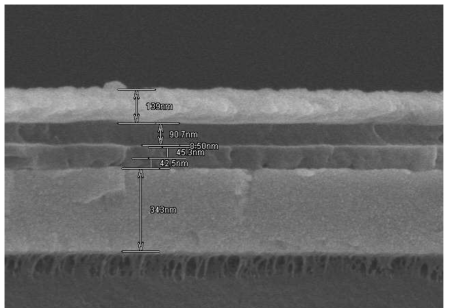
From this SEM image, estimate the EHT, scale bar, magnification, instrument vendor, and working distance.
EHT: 5 kV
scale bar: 500 nm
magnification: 70 K X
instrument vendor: Hitachi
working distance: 9.2 mm